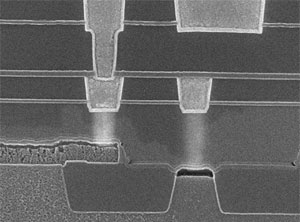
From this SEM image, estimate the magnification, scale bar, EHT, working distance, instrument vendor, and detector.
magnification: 50 K X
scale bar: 100 nm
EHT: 10 kV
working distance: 8.3 mm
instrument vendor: JEOL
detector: SEI